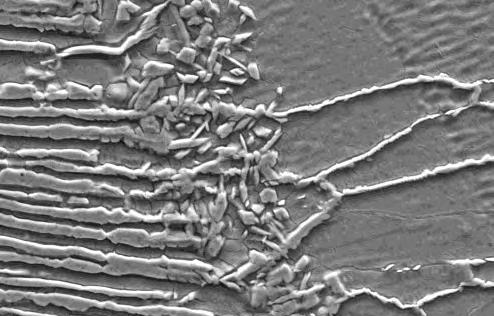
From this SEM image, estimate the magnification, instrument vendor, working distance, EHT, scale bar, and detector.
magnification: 3 K X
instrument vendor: Zeiss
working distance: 10 mm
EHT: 20 kV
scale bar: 10000 nm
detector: SE1